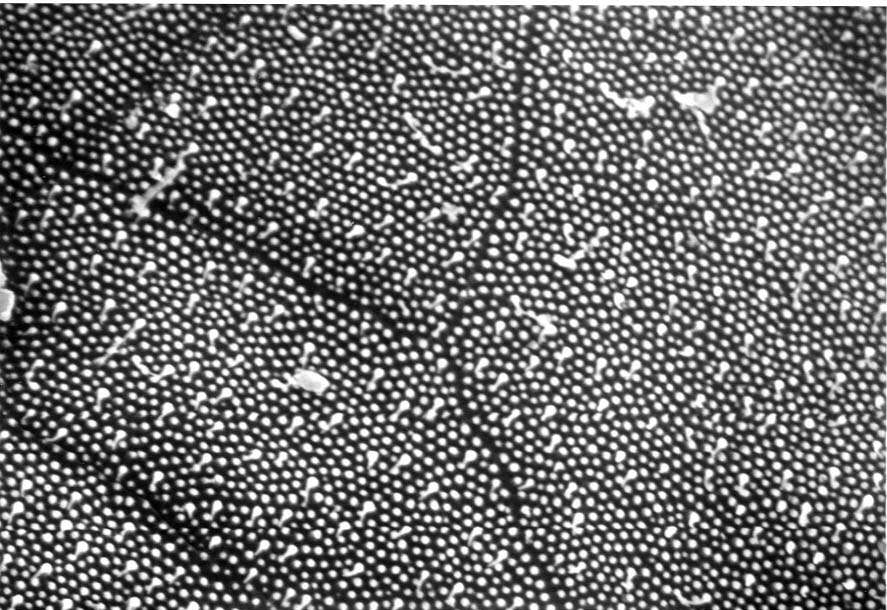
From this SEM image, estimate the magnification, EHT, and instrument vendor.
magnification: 6 K X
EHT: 20 kV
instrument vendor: JEOL